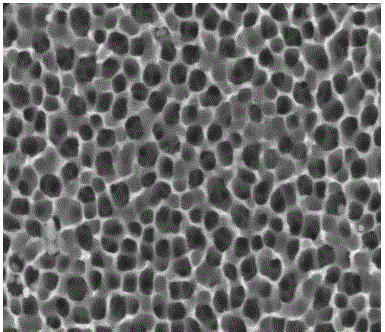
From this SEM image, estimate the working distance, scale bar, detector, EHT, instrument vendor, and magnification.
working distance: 11.3 mm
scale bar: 500 nm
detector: SE(M)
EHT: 5 kV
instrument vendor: Hitachi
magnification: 100 K X